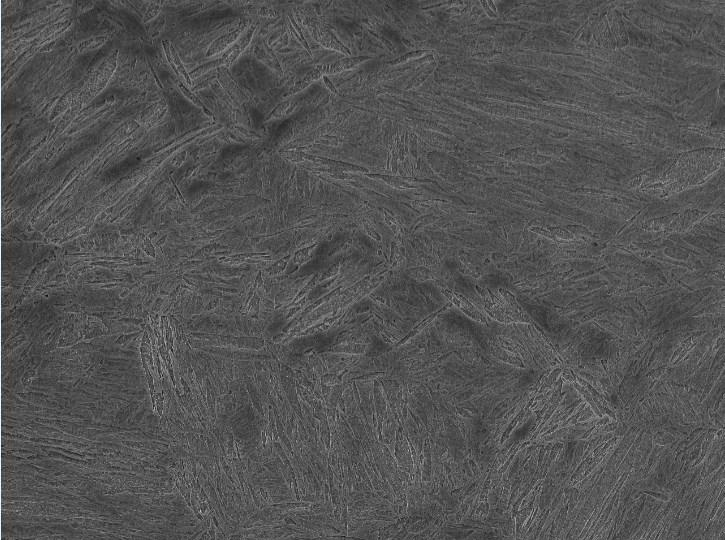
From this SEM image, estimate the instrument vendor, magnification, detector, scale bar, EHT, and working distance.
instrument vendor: JEOL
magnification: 2.2 K X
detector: SEI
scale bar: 10000 nm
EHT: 15 kV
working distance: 10.3 mm